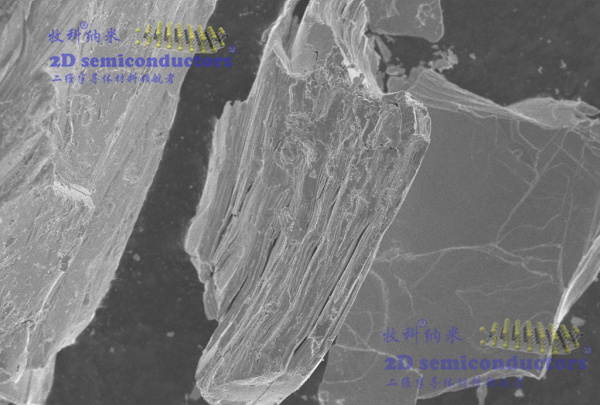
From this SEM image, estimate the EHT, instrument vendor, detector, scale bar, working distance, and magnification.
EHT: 5 kV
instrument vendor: Zeiss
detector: InLens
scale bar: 10000 nm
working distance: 7.5 mm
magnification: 1 K X